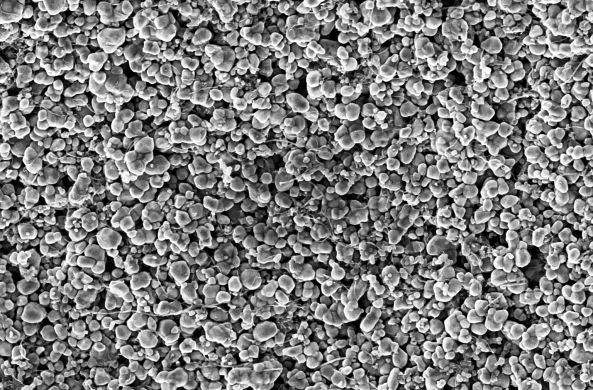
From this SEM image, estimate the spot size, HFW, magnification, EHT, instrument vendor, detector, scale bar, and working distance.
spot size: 5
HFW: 6.35 µm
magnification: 20 K X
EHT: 2 kV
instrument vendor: Thermo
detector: T2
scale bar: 1000 nm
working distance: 3.0468 mm